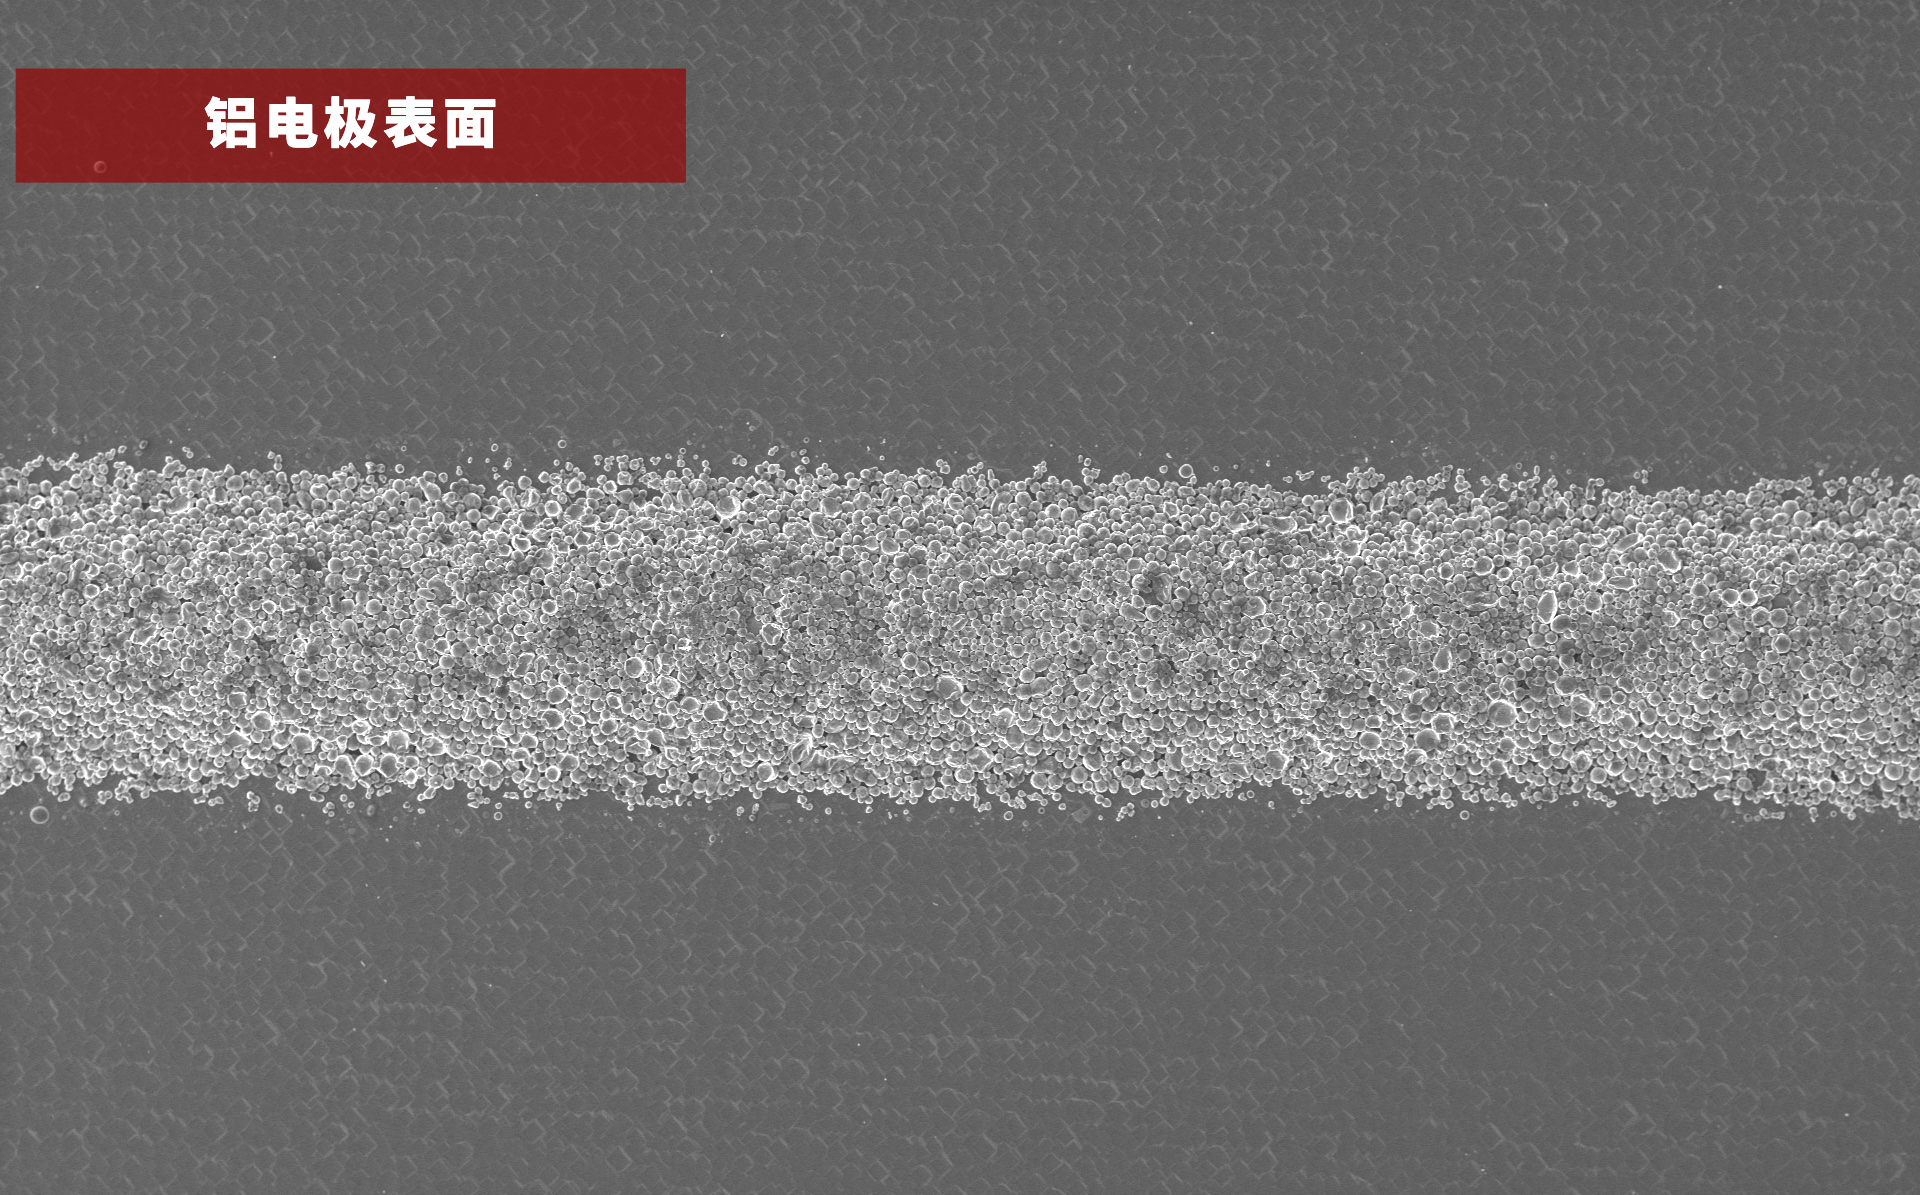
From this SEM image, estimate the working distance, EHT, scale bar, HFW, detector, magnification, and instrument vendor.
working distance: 6.979 mm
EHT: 10 kV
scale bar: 300000 nm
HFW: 1040 µm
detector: SED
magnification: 0.5 K X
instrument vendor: other